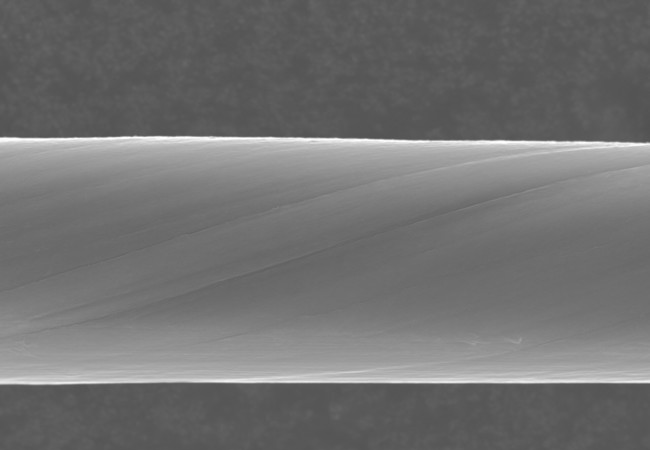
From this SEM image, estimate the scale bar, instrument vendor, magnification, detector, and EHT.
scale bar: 30000 nm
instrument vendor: Hitachi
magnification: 1.5 K X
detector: SE(M)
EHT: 20 kV